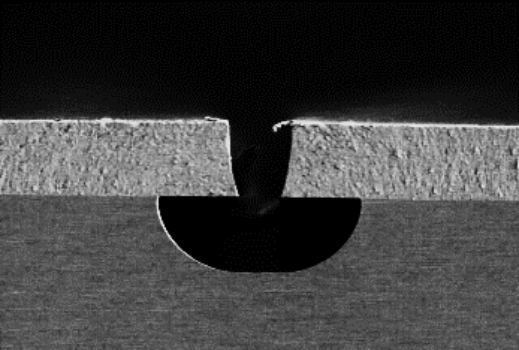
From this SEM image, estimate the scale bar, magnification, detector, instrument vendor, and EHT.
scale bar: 50000 nm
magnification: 1 K X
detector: SE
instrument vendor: Hitachi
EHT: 1 kV